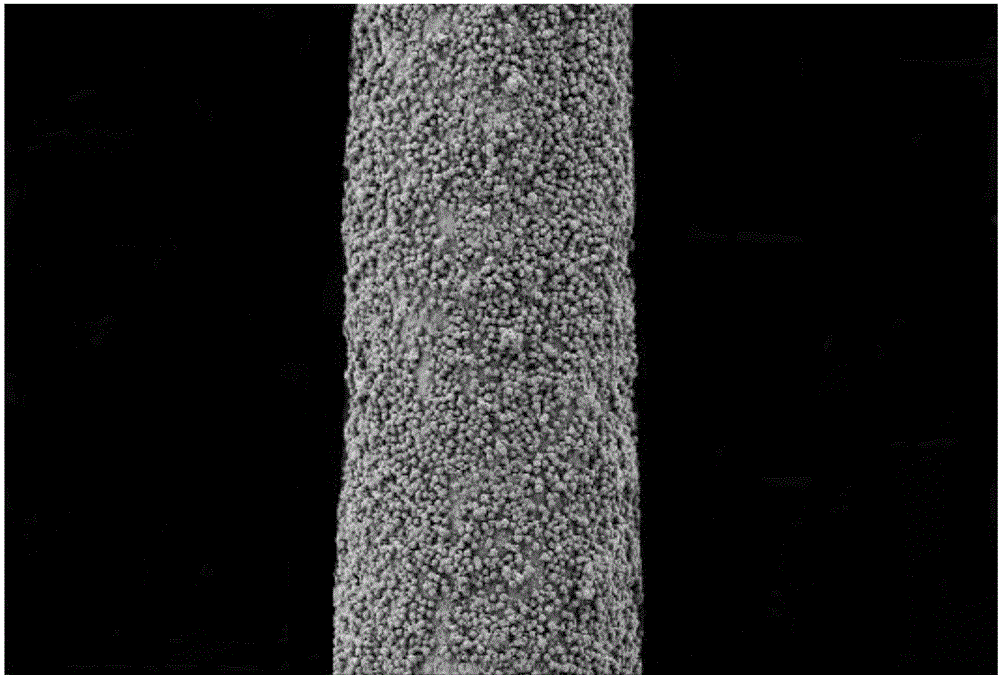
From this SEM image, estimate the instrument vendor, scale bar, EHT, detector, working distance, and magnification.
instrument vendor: Zeiss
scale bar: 20000 nm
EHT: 5 kV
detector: SE2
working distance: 7 mm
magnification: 0.25 K X